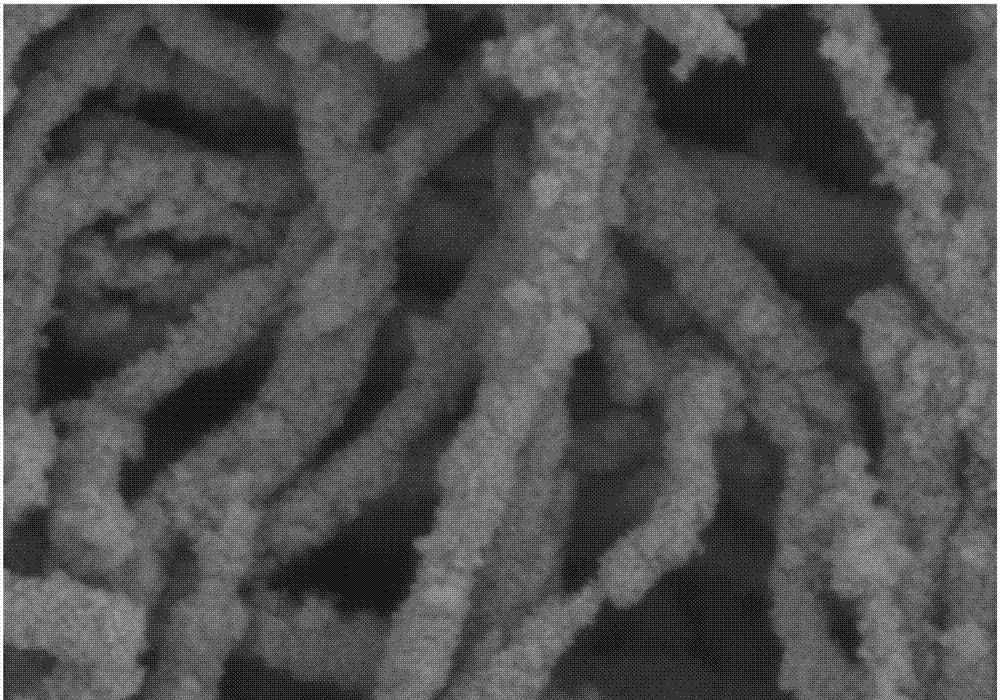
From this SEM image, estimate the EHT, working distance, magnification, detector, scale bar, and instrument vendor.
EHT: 3 kV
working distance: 9.6 mm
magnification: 50 K X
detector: SE(M)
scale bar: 1000 nm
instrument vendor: Hitachi